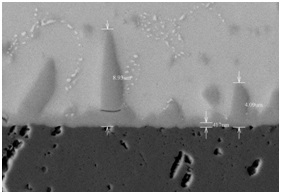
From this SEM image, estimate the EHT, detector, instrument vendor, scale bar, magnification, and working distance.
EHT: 15 kV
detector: BSE3D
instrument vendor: Hitachi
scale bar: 10000 nm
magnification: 5 K X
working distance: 9.1 mm